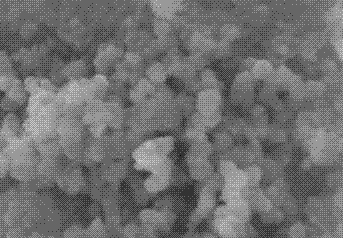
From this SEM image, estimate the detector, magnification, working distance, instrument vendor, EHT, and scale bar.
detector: SE(U)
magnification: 80 K X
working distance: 7.4 mm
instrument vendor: Hitachi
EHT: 5 kV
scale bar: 500 nm